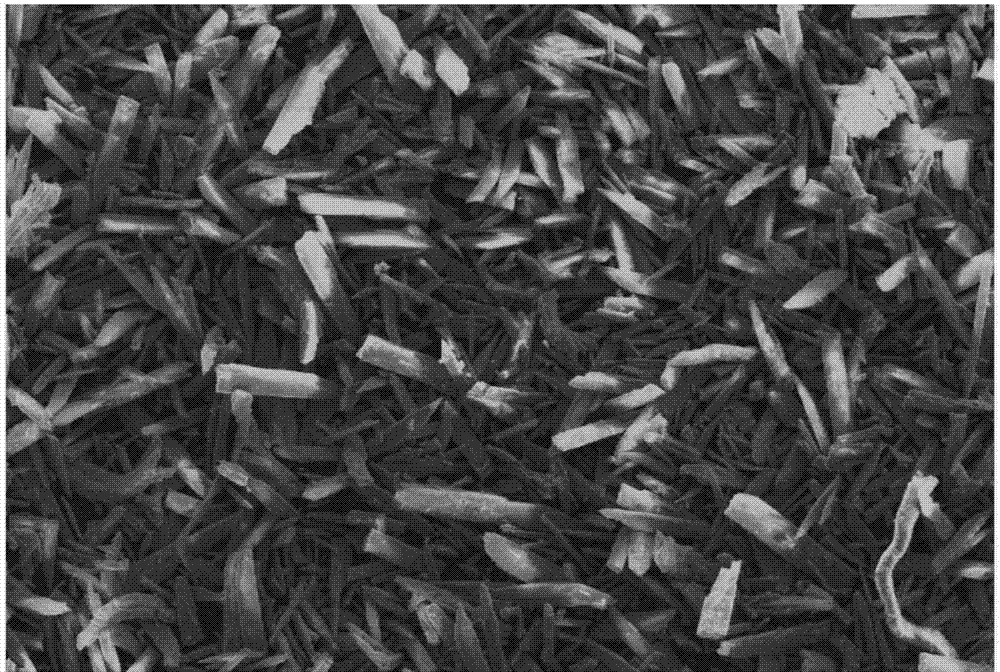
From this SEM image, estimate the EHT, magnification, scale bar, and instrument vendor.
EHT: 25 kV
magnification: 0.1 K X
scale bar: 100000 nm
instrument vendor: other